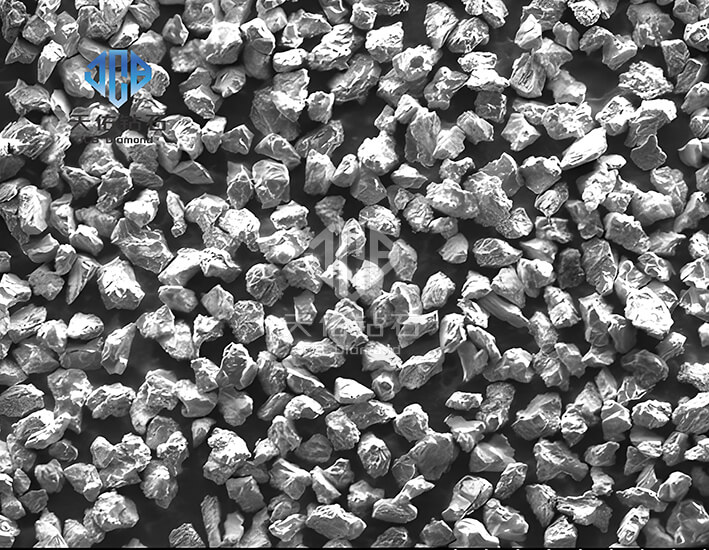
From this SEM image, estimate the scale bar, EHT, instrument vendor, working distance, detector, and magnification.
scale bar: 50000 nm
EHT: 5 kV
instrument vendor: Hitachi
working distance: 9.8 mm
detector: SE(M)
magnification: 1 K X